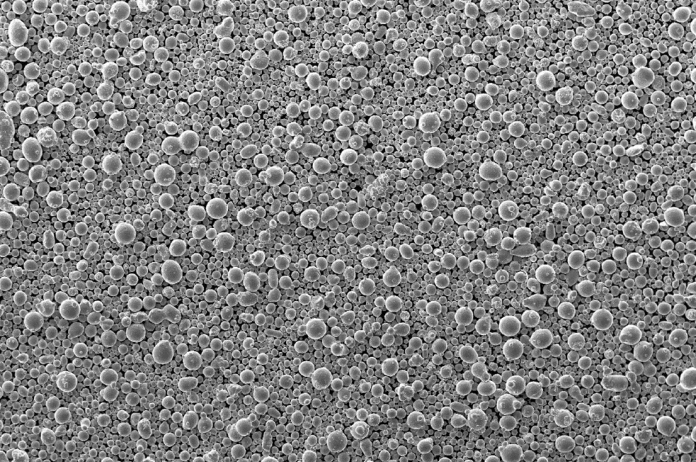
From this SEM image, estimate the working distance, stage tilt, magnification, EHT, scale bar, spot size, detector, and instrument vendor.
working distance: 9.8 mm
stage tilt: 0°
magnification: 0.05 K X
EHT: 20 kV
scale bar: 400000 nm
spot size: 4.7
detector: SE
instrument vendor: FEI